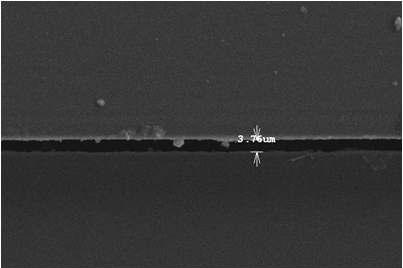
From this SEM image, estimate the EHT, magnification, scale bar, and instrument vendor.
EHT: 15 kV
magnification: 1 K X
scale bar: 50000 nm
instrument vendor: JEOL